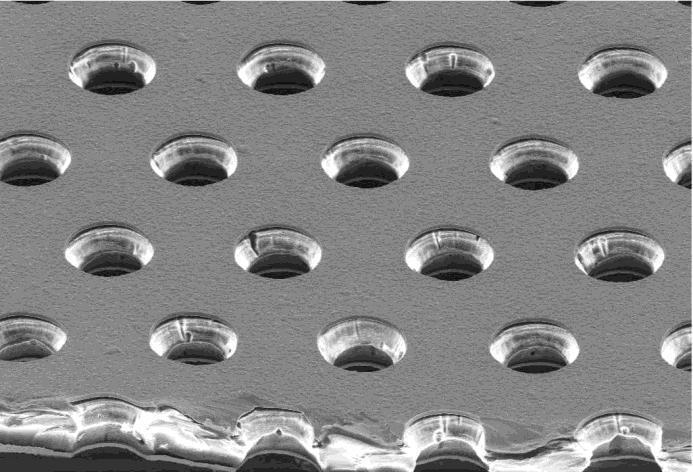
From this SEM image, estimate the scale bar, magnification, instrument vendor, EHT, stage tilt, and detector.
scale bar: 30000 nm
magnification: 0.2 K X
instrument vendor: Zeiss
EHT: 15 kV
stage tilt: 52°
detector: SE1